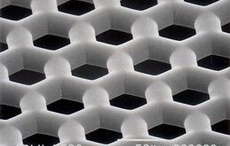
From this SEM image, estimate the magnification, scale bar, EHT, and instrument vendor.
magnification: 0.5 K X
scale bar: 50000 nm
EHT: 20 kV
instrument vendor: JEOL